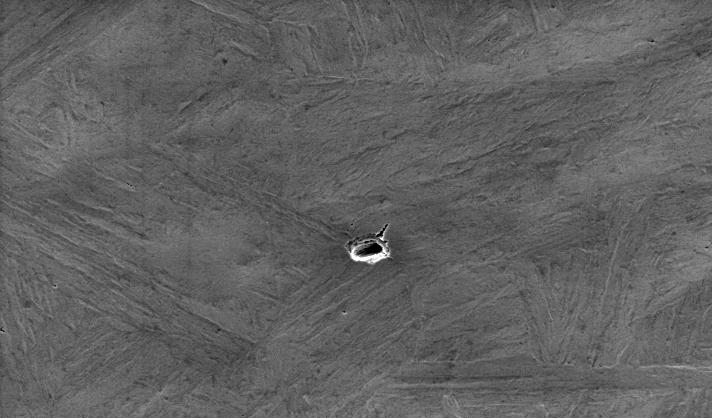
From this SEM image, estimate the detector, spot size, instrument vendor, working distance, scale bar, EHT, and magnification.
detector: SE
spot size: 5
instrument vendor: FEI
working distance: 9.7 mm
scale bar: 50000 nm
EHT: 20 kV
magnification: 0.5 K X